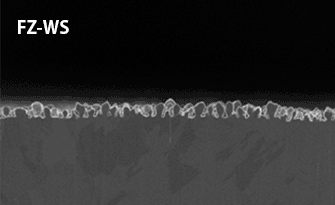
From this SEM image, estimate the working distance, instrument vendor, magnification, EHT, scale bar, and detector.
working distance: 3.3 mm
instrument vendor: Hitachi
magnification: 10 K X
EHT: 3 kV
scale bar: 5000 nm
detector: SE(U)